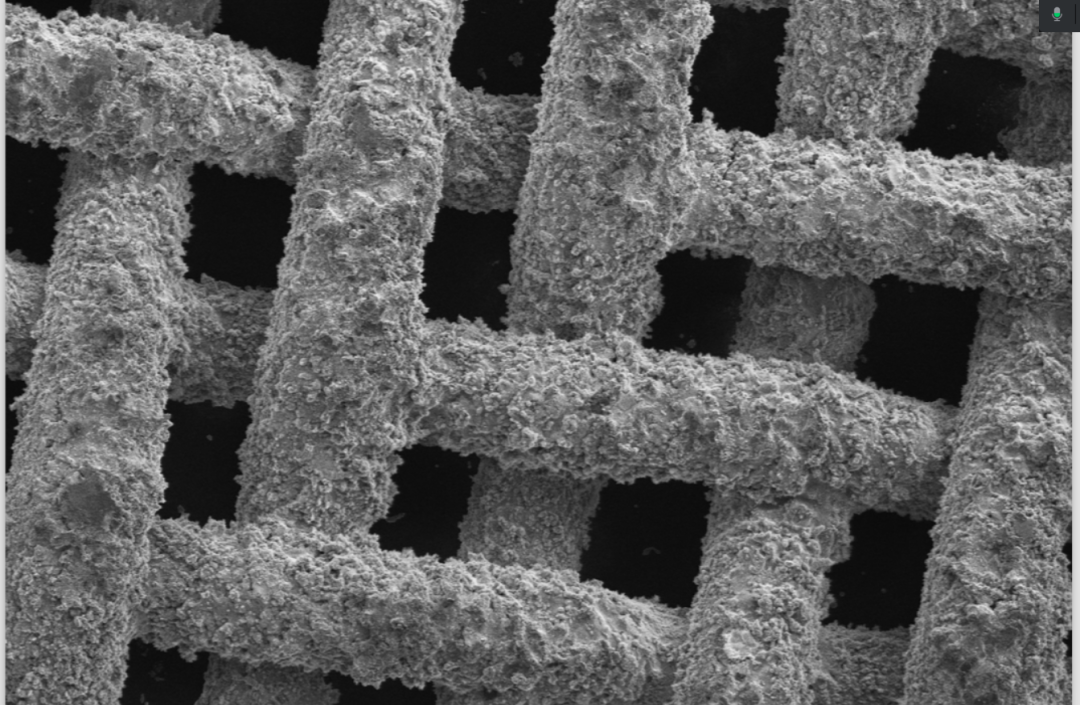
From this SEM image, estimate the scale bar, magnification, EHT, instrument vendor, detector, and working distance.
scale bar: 1000000 nm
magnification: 0.05 K X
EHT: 5 kV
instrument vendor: Hitachi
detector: SE(M)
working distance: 15.5 mm